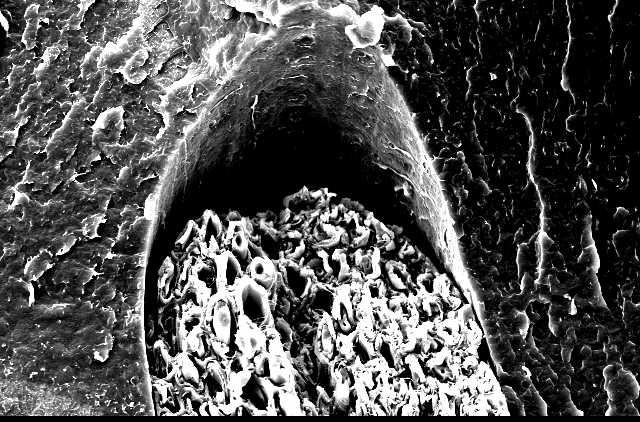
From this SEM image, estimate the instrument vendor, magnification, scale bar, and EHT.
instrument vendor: JEOL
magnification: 0.5 K X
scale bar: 50000 nm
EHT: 15 kV